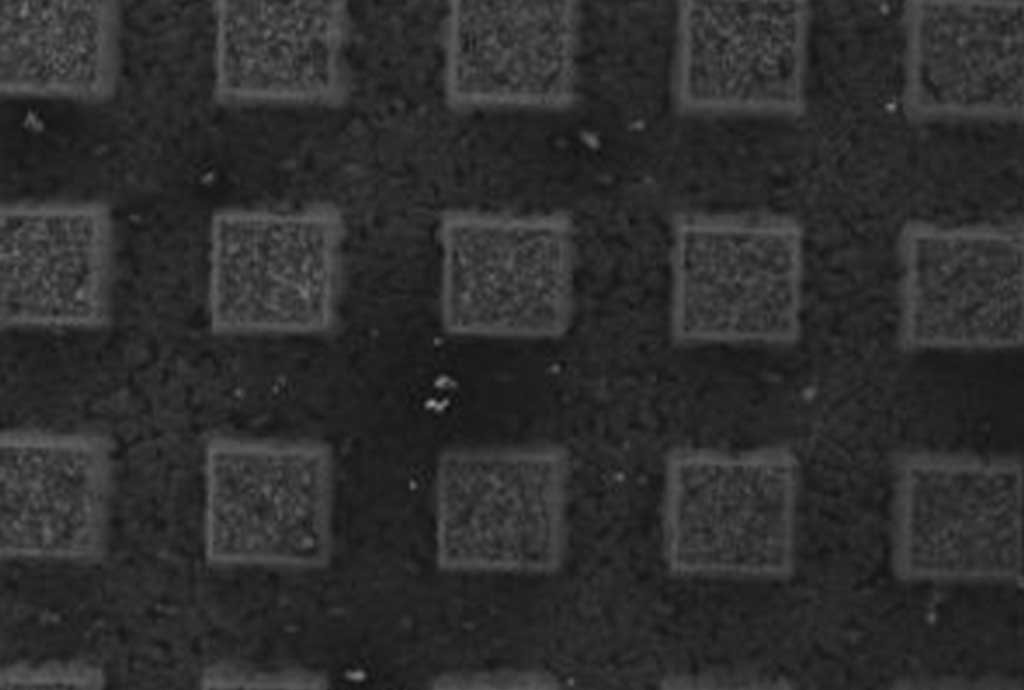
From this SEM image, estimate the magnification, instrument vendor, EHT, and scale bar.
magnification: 0.025 K X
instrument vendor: JEOL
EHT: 20 kV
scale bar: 1000000 nm